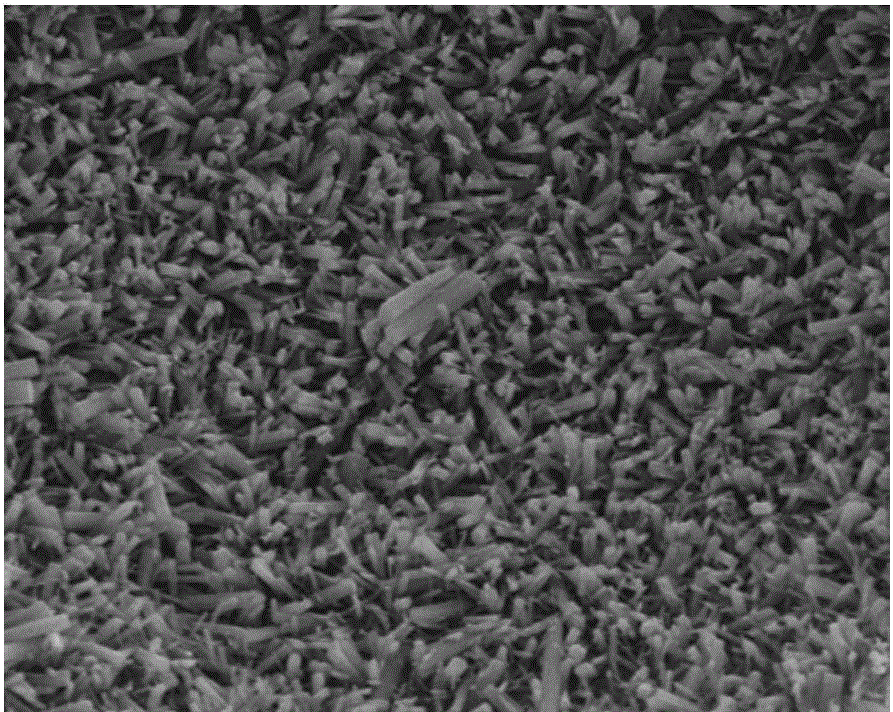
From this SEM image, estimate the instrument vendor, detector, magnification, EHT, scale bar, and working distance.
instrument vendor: FEI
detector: ETD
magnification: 5 K X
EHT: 15 kV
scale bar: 20000 nm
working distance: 10.5 mm